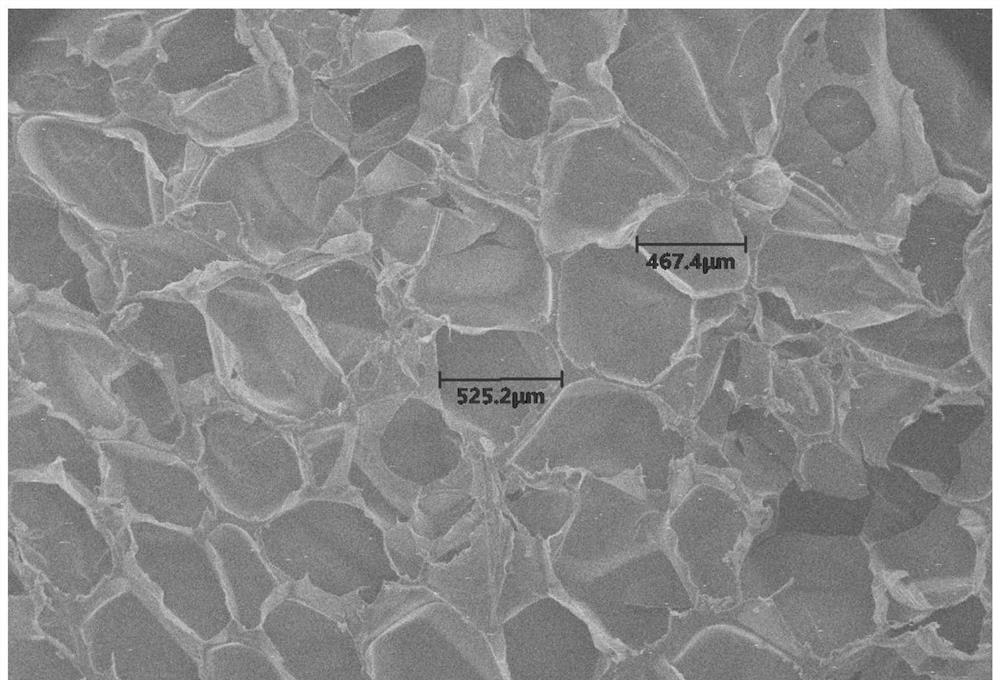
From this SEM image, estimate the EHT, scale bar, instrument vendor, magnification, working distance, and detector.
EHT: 20 kV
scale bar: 800000 nm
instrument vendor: other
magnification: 0.03 K X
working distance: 12.8 mm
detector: SE